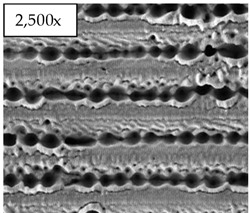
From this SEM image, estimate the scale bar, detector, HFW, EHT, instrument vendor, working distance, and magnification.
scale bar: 40000 nm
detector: ETD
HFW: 119 µm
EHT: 30 kV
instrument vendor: FEI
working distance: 7 mm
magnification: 2.5 K X